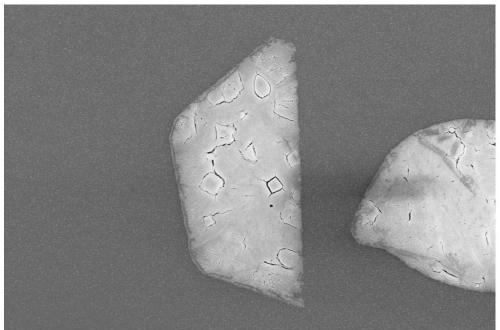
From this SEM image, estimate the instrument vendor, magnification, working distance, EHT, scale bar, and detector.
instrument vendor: Zeiss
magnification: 2.25 K X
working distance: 8.2 mm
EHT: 10 kV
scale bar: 2000 nm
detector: InLens